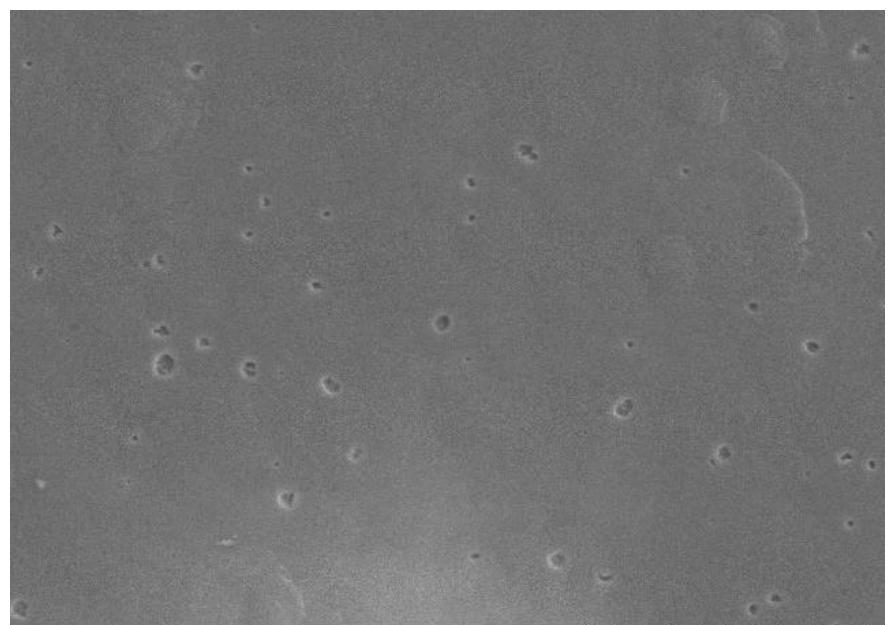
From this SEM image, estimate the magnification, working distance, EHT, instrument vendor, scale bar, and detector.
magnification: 2 K X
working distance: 12.2 mm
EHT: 10 kV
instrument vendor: Hitachi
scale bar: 20000 nm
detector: SE